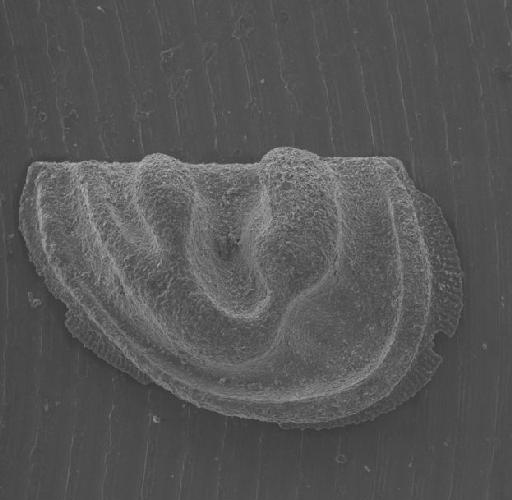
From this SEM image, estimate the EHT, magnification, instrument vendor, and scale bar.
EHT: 15 kV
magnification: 0.08 K X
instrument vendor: JEOL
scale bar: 380000 nm